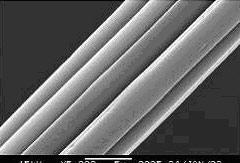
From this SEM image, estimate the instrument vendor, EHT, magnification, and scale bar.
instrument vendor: JEOL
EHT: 15 kV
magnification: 5 K X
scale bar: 5000 nm